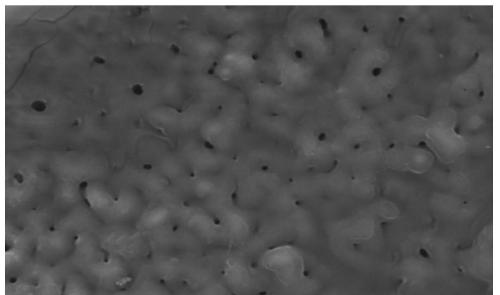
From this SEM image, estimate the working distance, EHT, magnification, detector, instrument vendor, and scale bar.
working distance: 10.7 mm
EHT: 15 kV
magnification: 2 K X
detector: LED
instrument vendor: JEOL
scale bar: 10000 nm